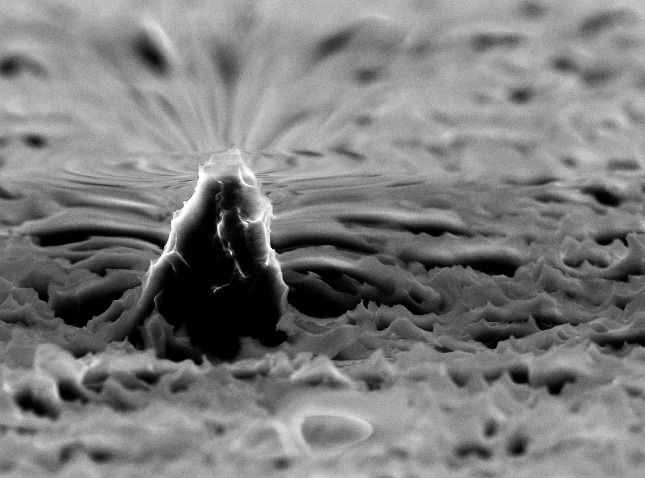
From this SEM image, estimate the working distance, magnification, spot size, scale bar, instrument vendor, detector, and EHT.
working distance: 11.1 mm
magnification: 4.031 K X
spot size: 3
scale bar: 5000 nm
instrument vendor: FEI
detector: SE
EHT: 10 kV